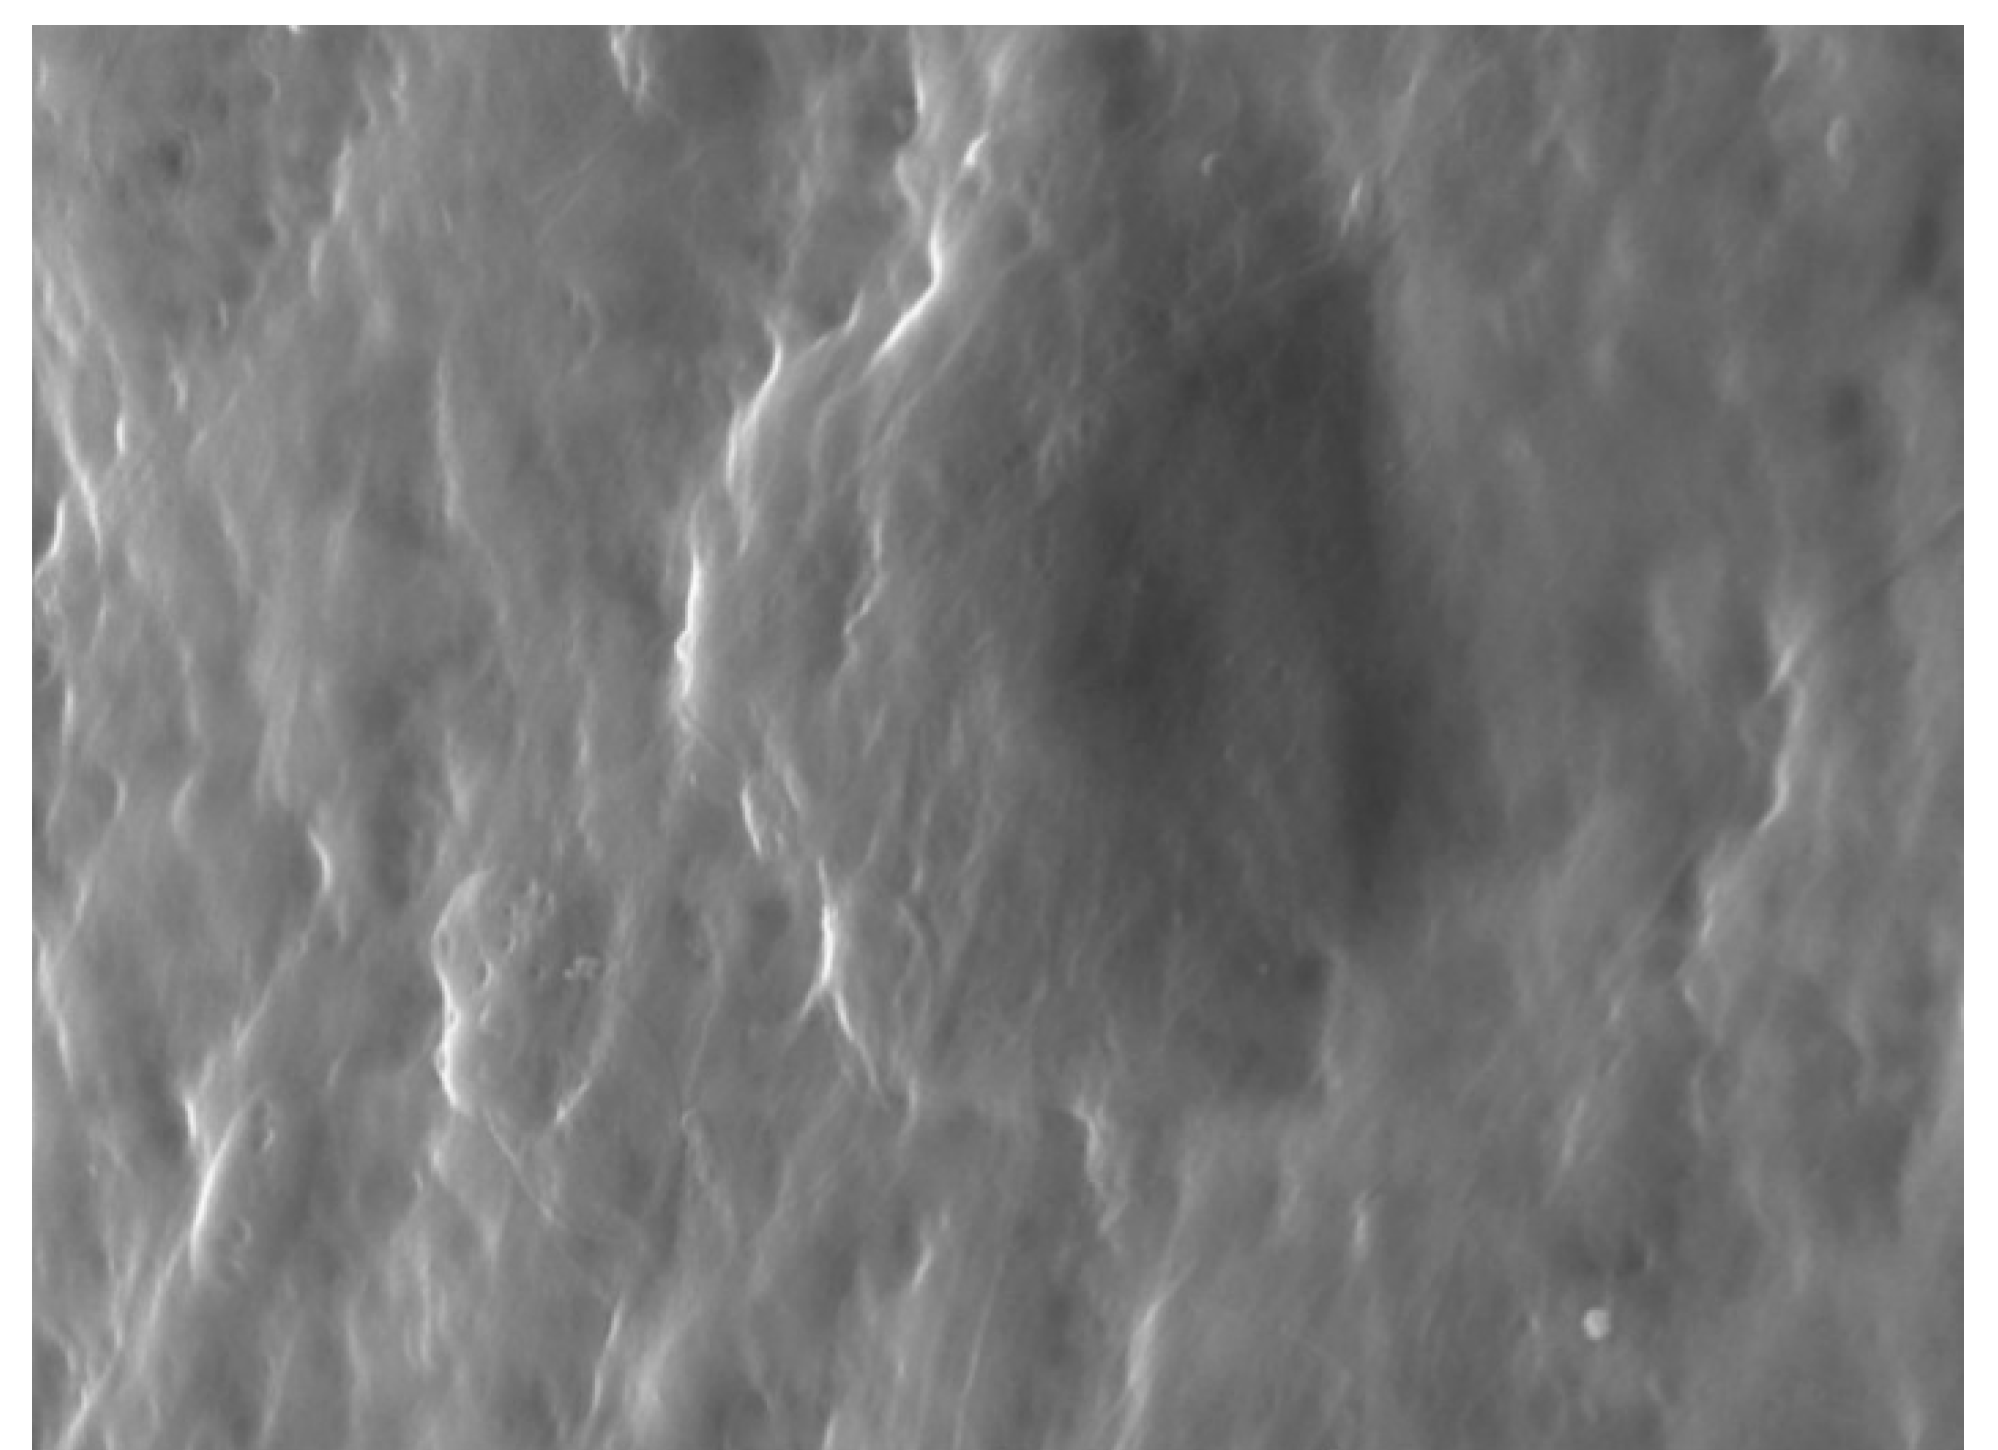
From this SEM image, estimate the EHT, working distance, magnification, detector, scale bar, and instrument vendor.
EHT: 20 kV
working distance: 15 mm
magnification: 5 K X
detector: SE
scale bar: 10000 nm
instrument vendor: Tescan